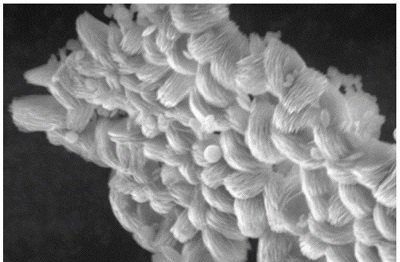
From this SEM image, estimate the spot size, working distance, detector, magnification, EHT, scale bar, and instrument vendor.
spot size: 5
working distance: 10.9 mm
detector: GSED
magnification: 8 K X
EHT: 20 kV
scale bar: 10000 nm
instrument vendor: FEI